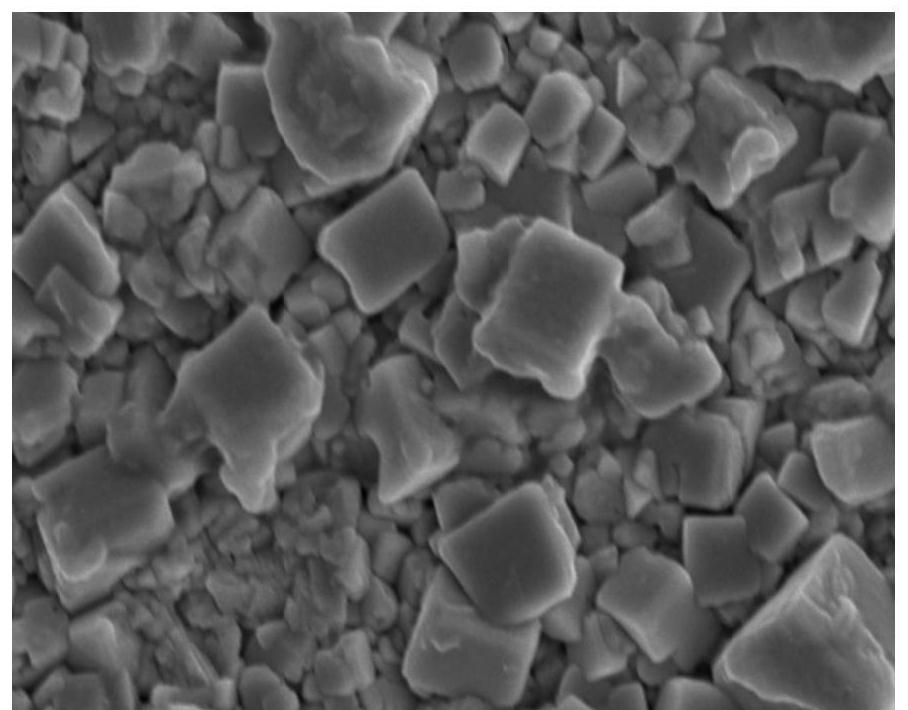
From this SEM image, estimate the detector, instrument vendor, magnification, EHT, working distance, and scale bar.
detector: SE(UL)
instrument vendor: Hitachi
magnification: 30 K X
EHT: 5 kV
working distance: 11.1 mm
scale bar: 1000 nm